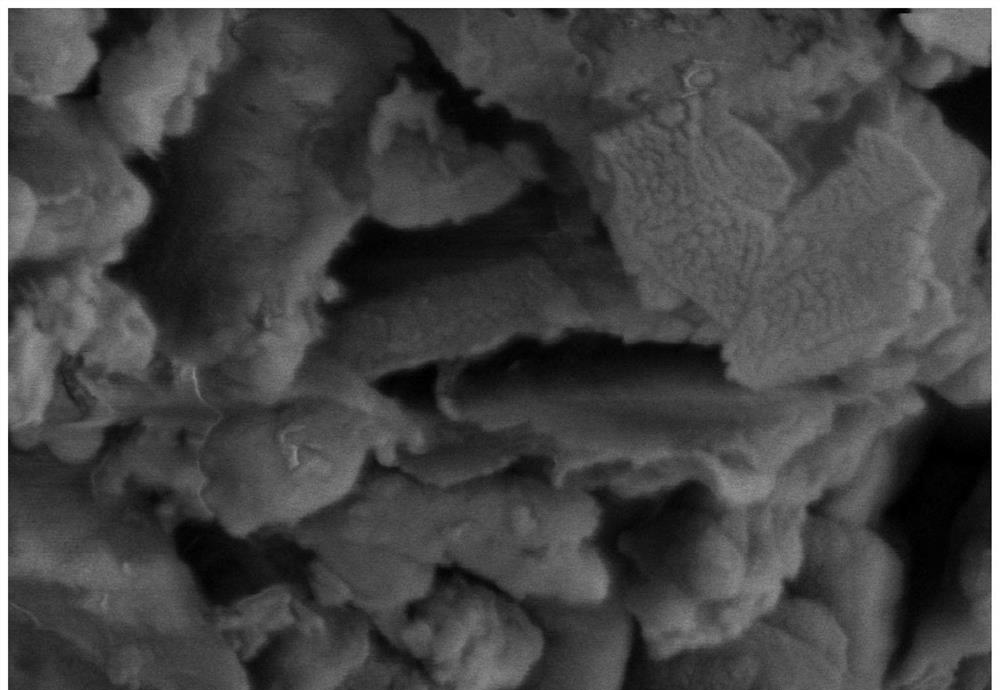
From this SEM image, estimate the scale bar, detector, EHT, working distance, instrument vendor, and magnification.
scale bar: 1000 nm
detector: SE(UL)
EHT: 3 kV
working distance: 13.3 mm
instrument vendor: Hitachi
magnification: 50 K X